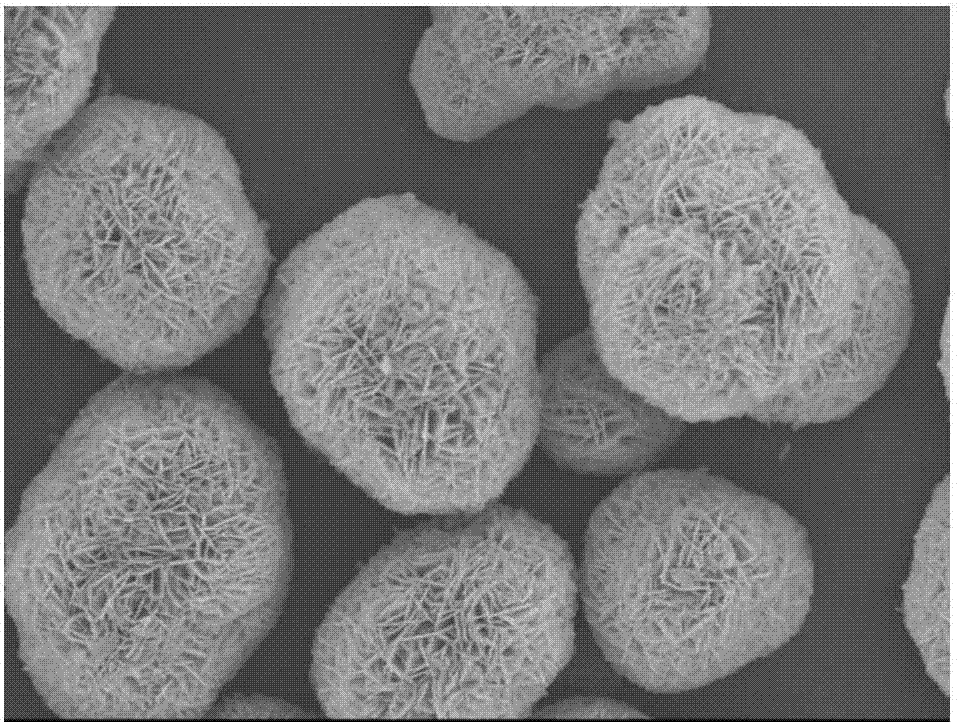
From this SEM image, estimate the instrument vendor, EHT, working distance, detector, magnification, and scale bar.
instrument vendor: JEOL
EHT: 10 kV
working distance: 7.6 mm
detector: SEI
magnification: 5 K X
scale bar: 1000 nm